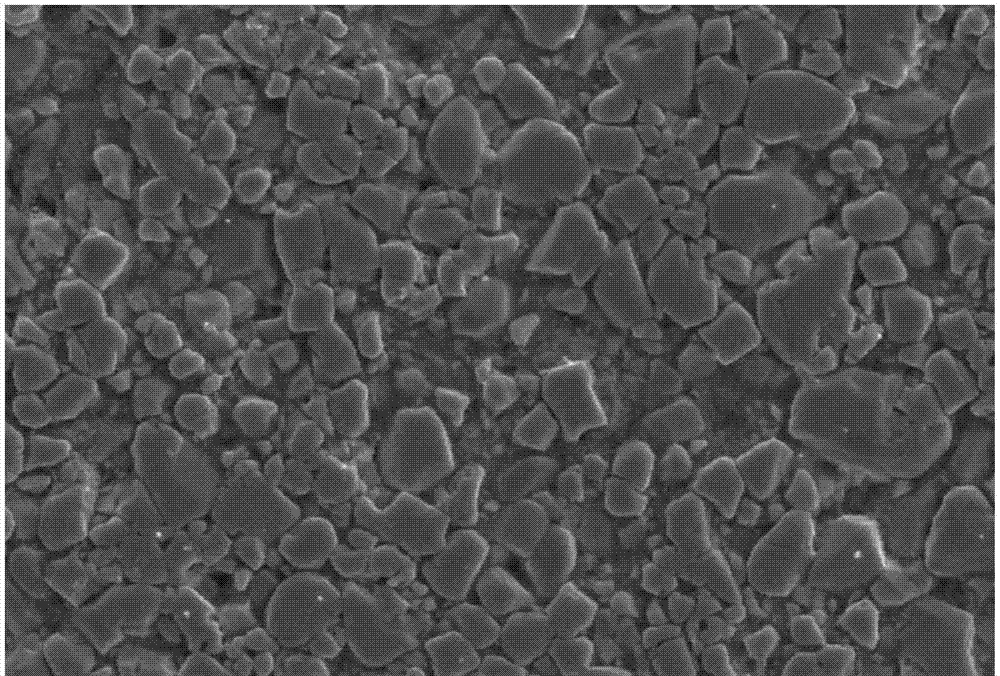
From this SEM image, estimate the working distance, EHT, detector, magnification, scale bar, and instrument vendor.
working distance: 10 mm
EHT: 20 kV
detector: SEI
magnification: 3 K X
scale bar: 5000 nm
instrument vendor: JEOL